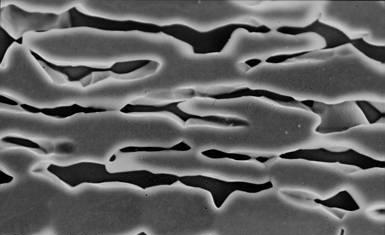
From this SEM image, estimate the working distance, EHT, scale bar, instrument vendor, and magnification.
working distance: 15 mm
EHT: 15 kV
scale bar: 1000 nm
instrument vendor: JEOL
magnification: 4.5 K X